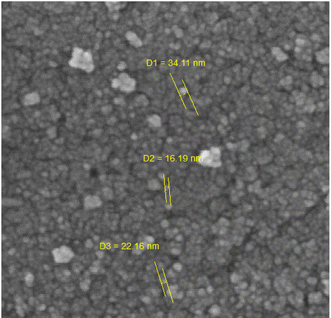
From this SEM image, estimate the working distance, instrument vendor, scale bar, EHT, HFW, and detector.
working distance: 16.58 mm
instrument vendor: Tescan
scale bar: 200 nm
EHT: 10 kV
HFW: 1.27 µm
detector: SE